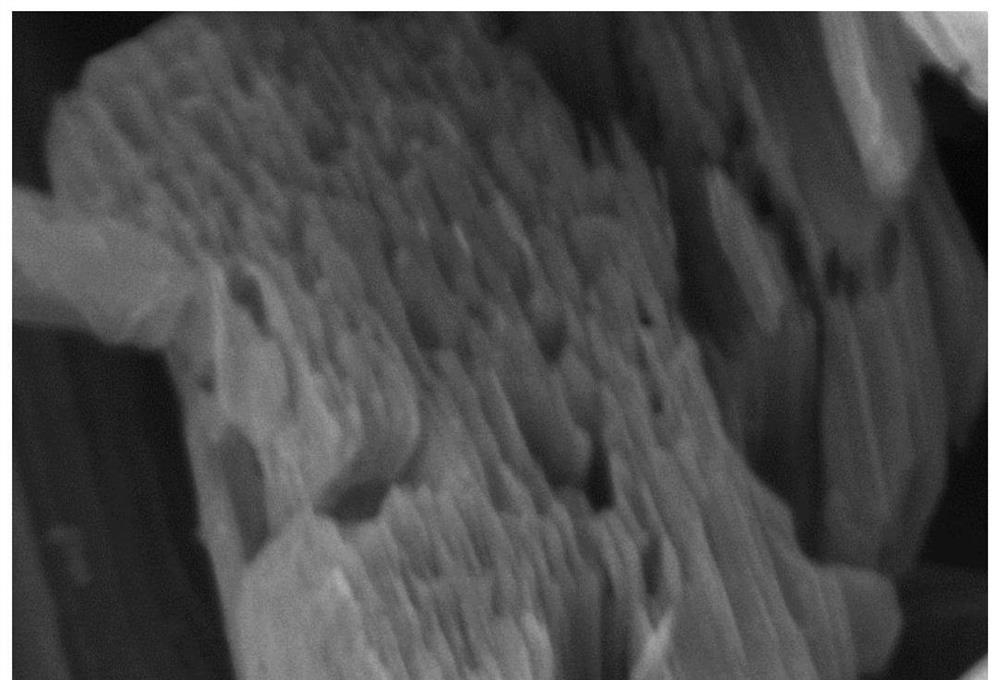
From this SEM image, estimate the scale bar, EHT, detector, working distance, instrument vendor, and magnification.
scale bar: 500 nm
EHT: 5 kV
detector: SE(UL)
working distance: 8.9 mm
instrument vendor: Hitachi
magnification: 100 K X